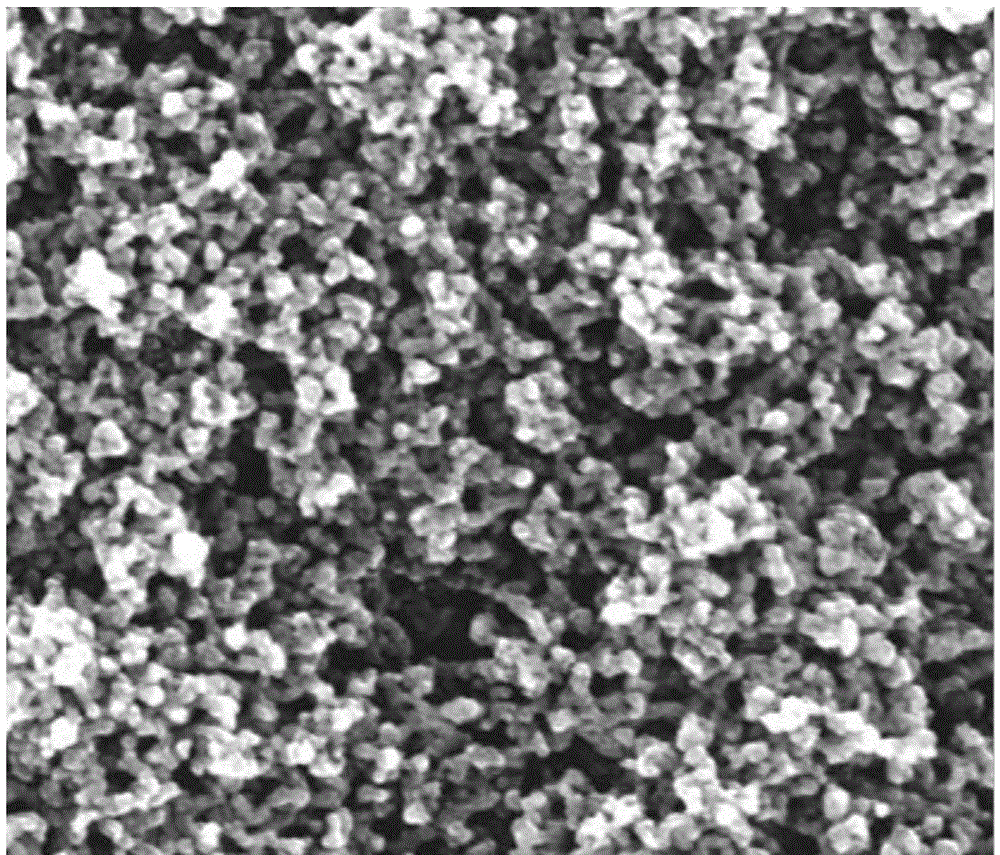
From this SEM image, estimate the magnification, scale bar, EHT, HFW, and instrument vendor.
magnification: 100 K X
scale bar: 500 nm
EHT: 10 kV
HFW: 1.49 µm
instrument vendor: FEI